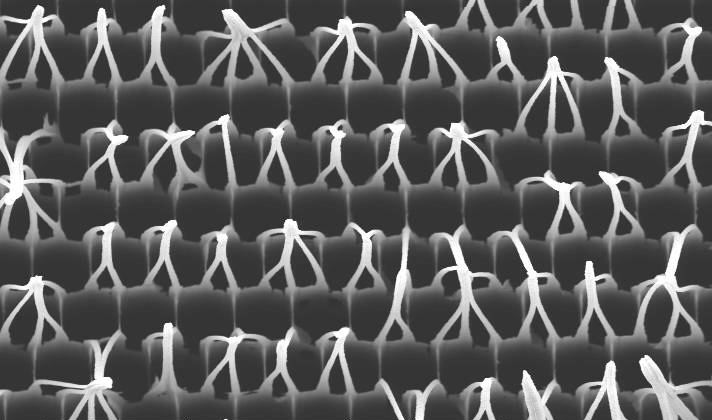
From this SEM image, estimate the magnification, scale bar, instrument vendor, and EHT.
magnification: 7.5 K X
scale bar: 10000 nm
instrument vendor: FEI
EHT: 7 kV